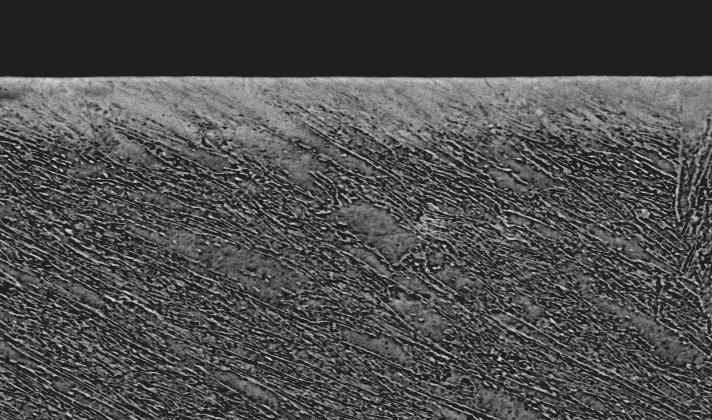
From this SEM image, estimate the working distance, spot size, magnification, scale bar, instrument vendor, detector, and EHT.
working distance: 12.3 mm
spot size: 5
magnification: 1.5 K X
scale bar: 20000 nm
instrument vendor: FEI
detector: BSE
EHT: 20 kV